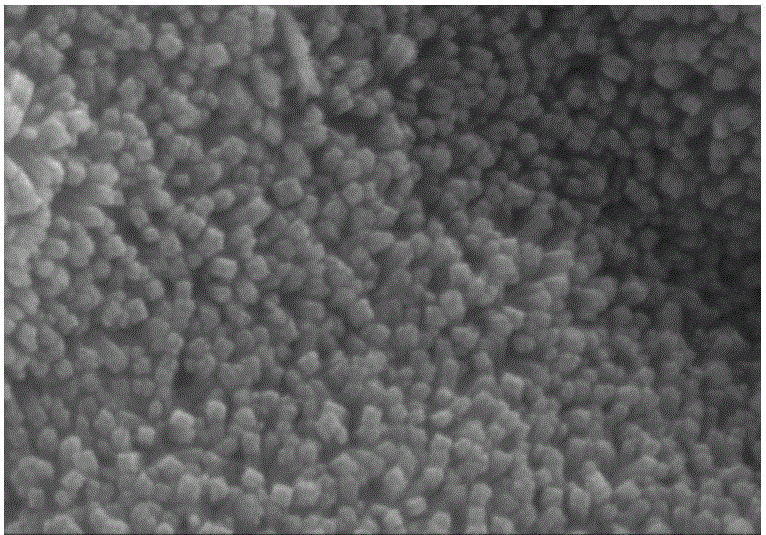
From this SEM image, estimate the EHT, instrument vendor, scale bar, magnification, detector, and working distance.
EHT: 20 kV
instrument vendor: Hitachi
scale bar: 500 nm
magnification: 100 K X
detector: SE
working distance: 8.7 mm